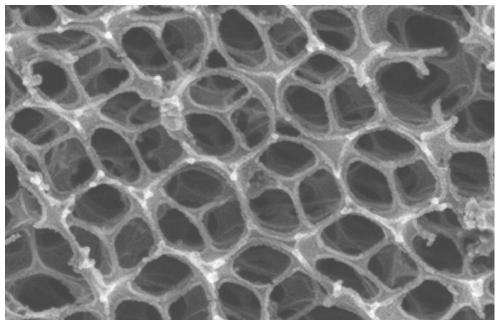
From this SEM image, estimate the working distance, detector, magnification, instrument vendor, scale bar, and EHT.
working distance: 8.5 mm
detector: SE(U)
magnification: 120 K X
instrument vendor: Hitachi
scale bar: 400 nm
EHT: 10 kV